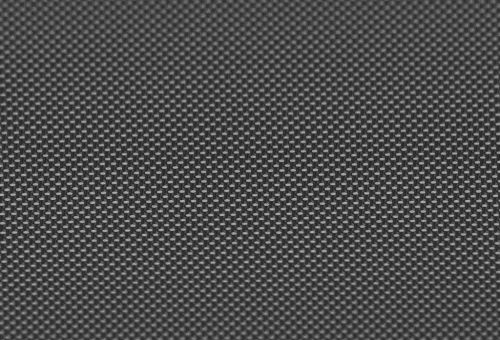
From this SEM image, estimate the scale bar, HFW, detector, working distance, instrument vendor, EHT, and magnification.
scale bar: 1000 nm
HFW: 50.21 µm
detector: SE2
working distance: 6.6 mm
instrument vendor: Zeiss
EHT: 2 kV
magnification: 5.98 K X